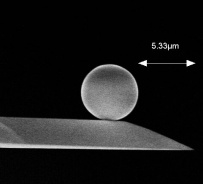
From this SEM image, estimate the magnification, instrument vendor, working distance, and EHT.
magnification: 5.06 K X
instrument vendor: Hitachi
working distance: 15 mm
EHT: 10 kV